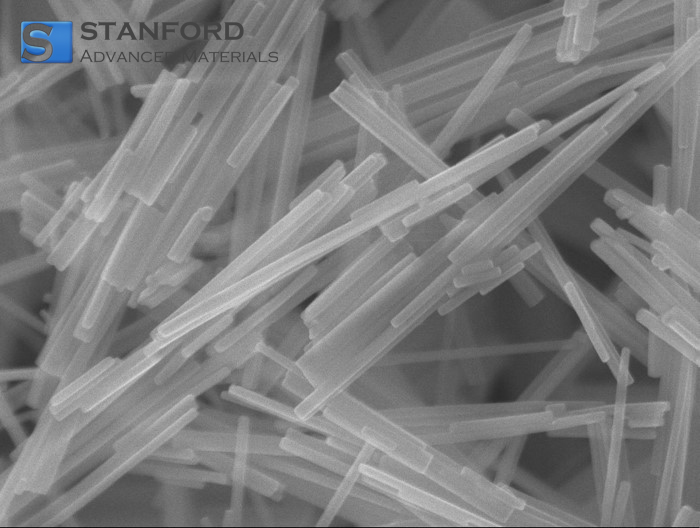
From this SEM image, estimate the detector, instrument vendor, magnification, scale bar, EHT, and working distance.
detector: SEI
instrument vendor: JEOL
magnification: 60 K X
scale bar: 100 nm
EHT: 10 kV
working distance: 3 mm